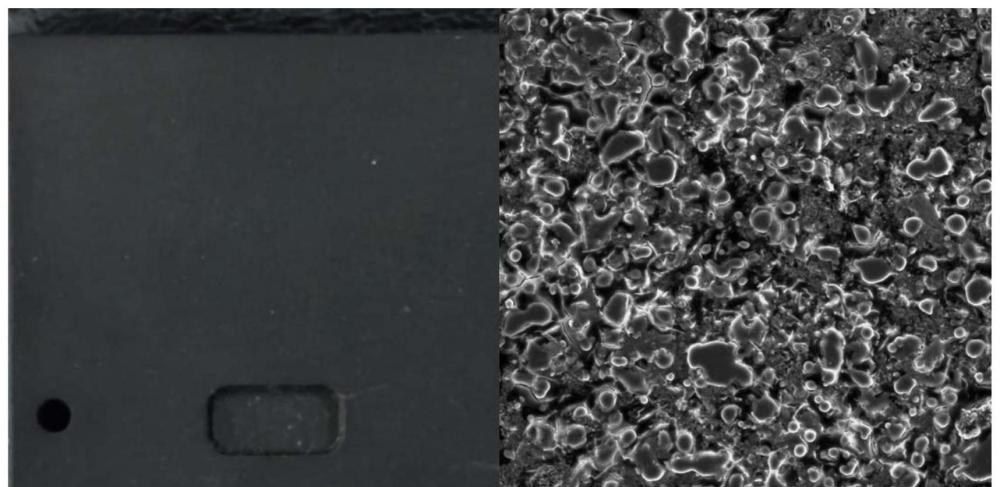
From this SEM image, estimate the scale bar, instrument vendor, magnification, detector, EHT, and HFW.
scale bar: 30000 nm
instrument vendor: Thermo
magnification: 1 K X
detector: SED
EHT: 10 kV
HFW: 125 µm